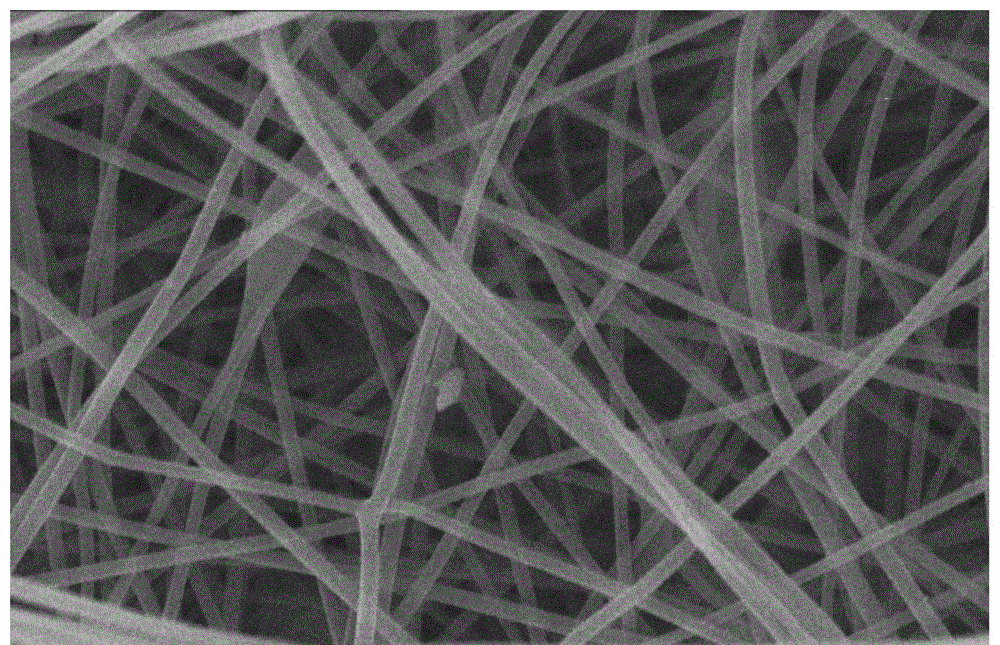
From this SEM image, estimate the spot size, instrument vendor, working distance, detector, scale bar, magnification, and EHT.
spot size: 9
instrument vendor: other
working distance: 6.4 mm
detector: SEI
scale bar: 1000 nm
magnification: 10 K X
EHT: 20 kV